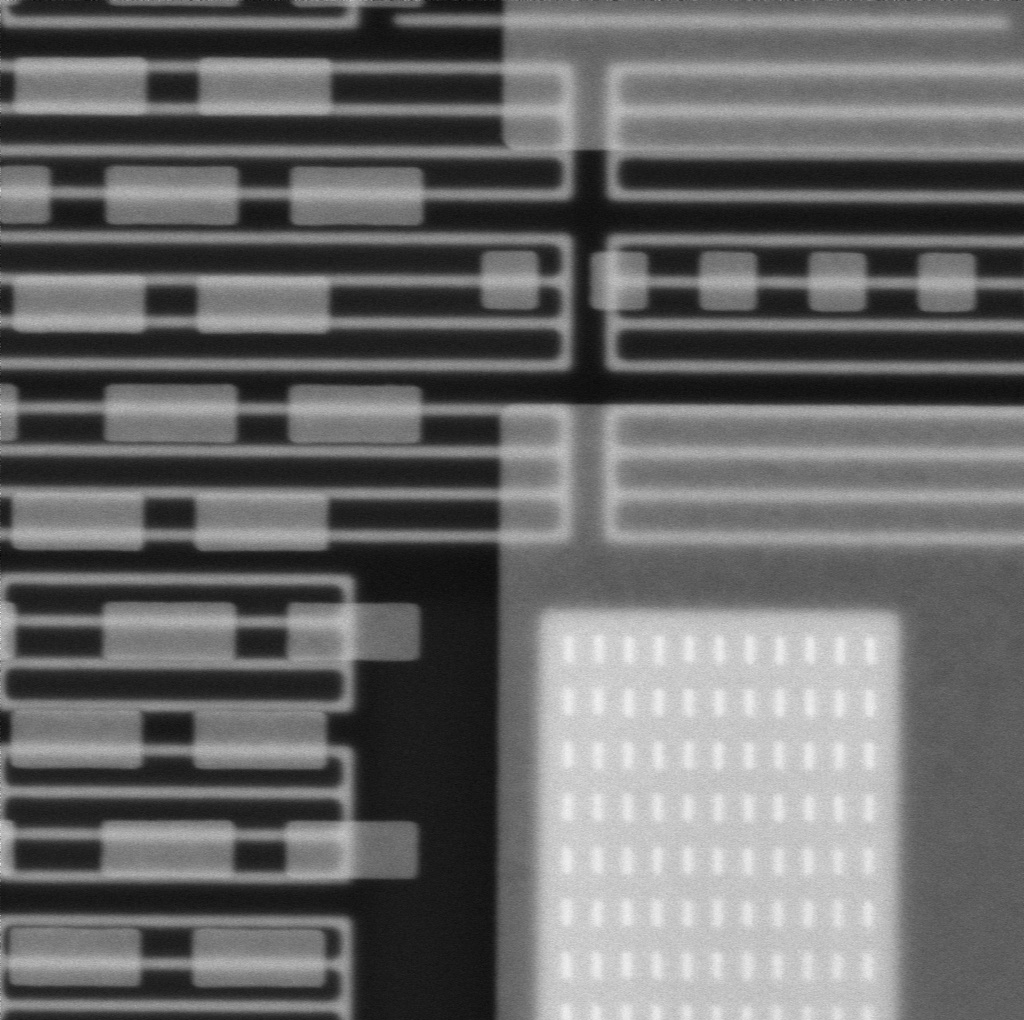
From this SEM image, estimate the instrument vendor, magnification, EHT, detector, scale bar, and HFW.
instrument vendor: other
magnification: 50 K X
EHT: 15 kV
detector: BSD Full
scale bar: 1000 nm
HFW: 5.37 µm